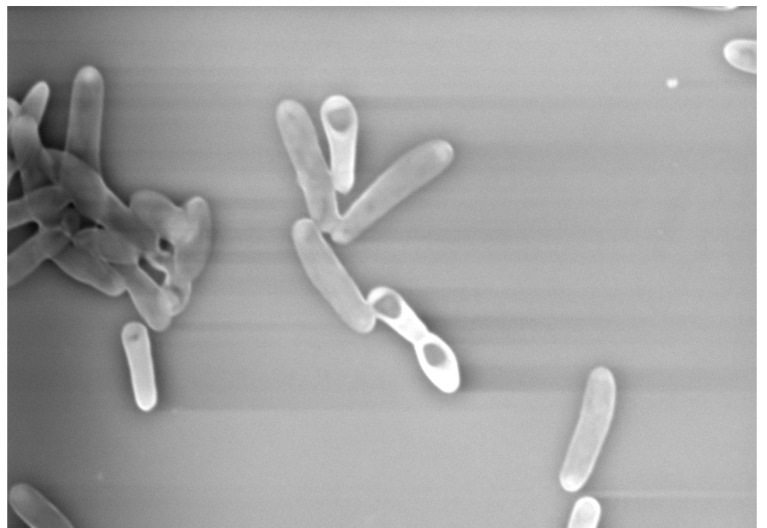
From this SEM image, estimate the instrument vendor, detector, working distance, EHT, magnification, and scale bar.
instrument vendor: Hitachi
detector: BSE M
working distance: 7 mm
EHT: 15 kV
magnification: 5 K X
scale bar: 10000 nm